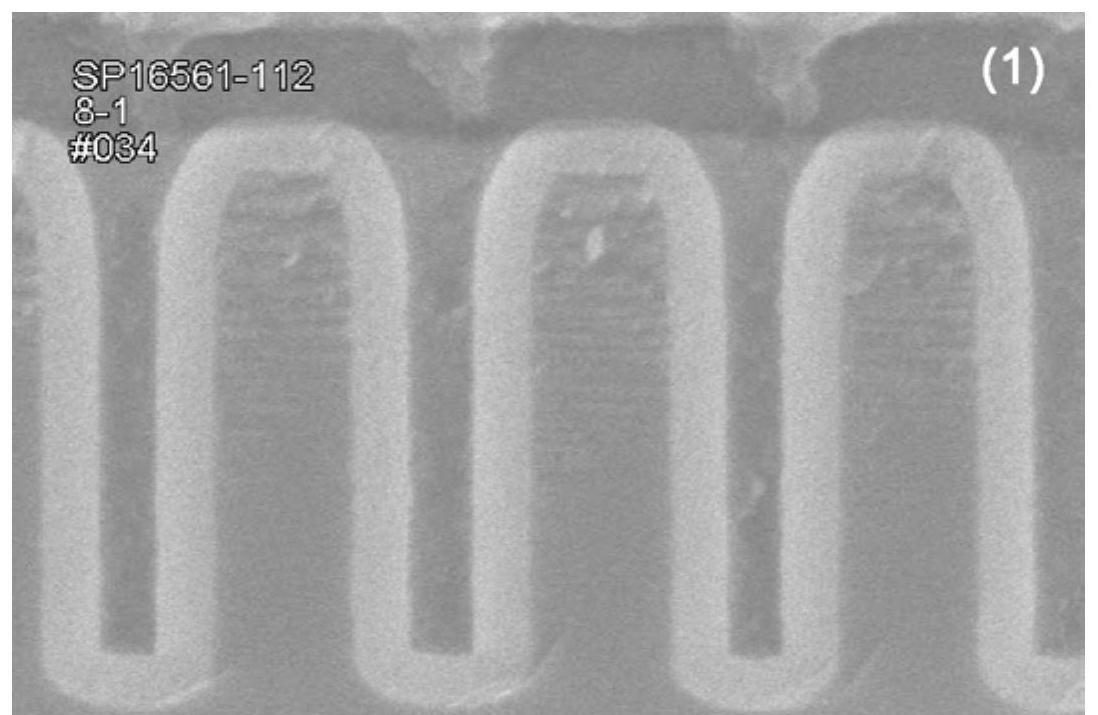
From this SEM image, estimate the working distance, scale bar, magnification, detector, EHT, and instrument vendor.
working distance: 3.5 mm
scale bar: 500 nm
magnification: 100 K X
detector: SE(U)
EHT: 2 kV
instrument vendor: Hitachi